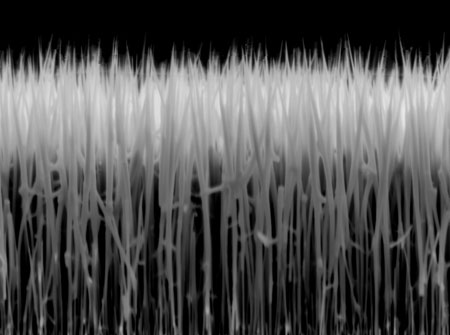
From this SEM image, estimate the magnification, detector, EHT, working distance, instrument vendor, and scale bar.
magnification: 16 K X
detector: SEI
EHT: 5 kV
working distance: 6 mm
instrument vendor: JEOL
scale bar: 1000 nm